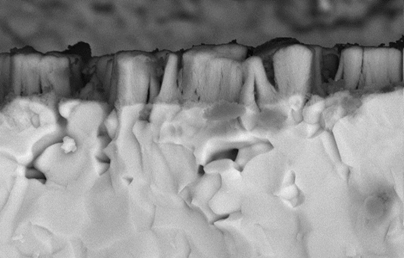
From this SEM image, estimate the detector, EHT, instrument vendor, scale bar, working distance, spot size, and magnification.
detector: BSE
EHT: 15 kV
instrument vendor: FEI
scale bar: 5000 nm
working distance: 14.1 mm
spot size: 3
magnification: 5 K X